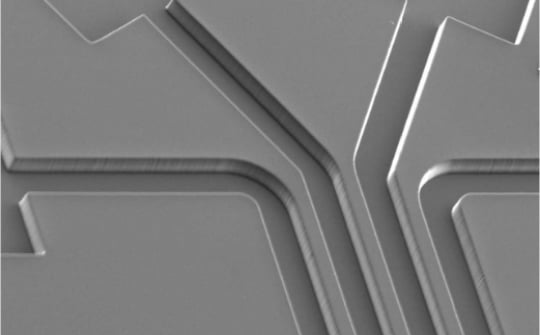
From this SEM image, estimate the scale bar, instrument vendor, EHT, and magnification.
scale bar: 200000 nm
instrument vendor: JEOL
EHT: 15 kV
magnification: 0.09 K X